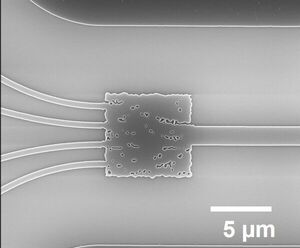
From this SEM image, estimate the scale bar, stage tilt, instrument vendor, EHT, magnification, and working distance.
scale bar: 5000 nm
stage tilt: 0°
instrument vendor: FEI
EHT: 19 kV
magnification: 5.743 K X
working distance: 5.2 mm